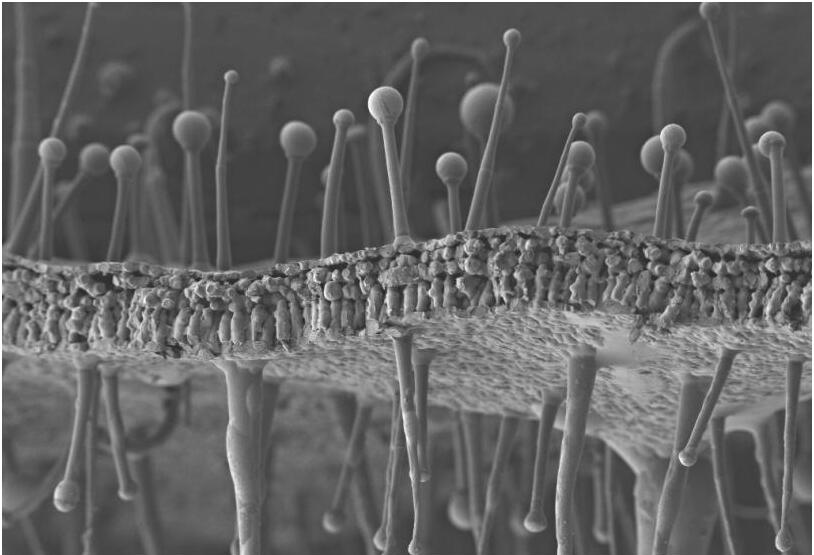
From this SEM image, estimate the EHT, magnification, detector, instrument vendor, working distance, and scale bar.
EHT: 2 kV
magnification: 0.081 K X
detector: SE2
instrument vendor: Zeiss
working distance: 5.3 mm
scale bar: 100000 nm